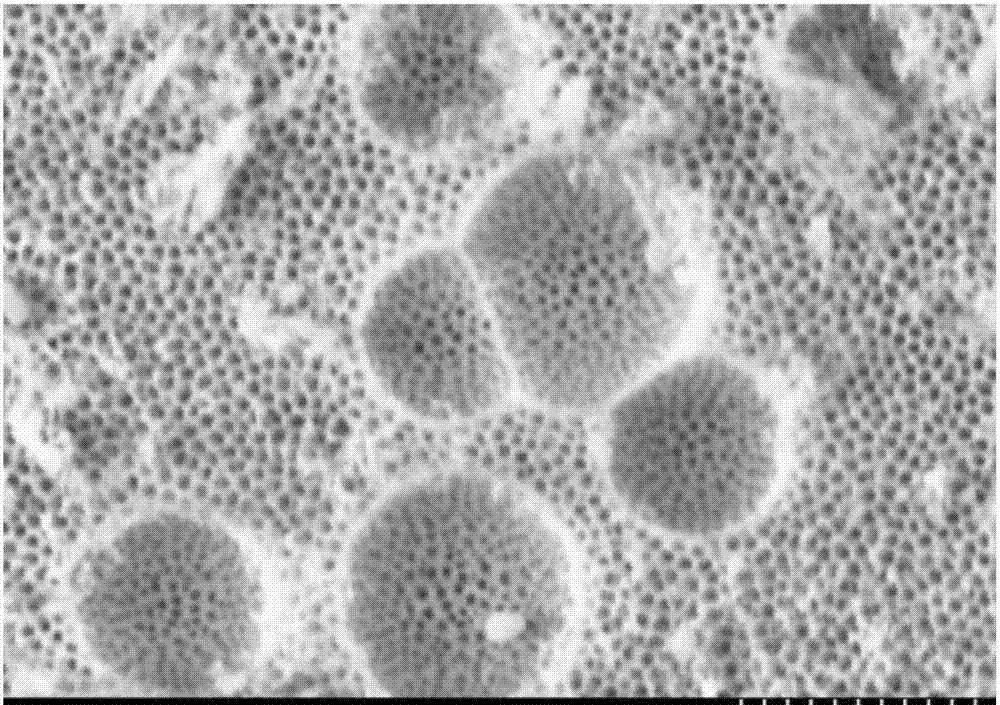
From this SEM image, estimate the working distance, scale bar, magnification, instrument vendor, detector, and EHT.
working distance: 8.7 mm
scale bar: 1000 nm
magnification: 30 K X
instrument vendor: Hitachi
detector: SE(M)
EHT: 5 kV